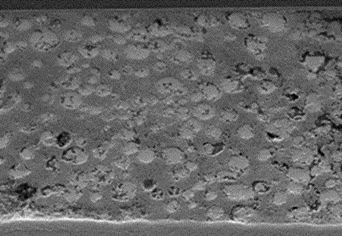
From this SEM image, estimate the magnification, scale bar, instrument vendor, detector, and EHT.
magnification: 2 K X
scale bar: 20000 nm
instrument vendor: Hitachi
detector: SE(M,LA100)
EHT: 10 kV